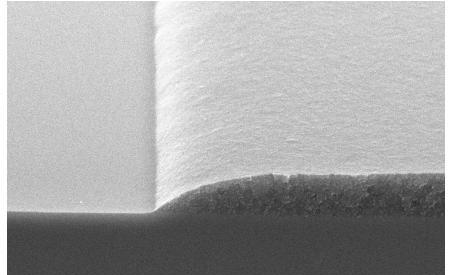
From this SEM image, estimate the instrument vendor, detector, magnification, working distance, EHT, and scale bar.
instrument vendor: JEOL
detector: SEI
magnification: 8 K X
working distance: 13 mm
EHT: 10 kV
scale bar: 2000 nm